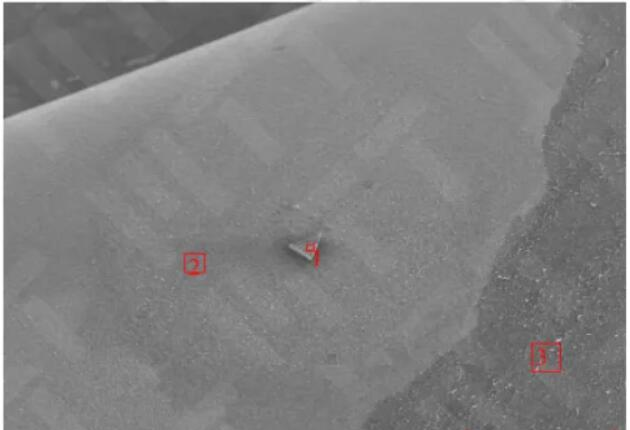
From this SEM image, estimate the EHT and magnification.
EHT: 15 kV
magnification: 0.06 K X